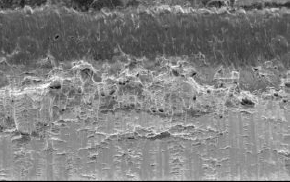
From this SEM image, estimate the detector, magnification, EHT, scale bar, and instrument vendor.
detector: SE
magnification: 0.54 K X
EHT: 15 kV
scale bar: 50000 nm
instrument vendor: FEI